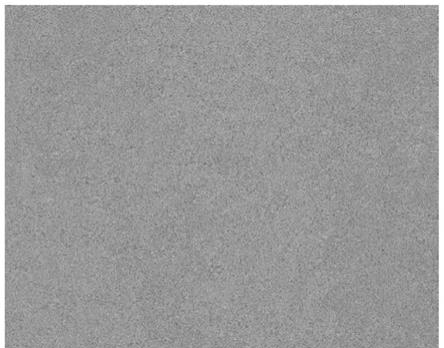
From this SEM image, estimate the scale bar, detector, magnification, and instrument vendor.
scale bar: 150000 nm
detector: BSD Full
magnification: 1 K X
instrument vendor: other